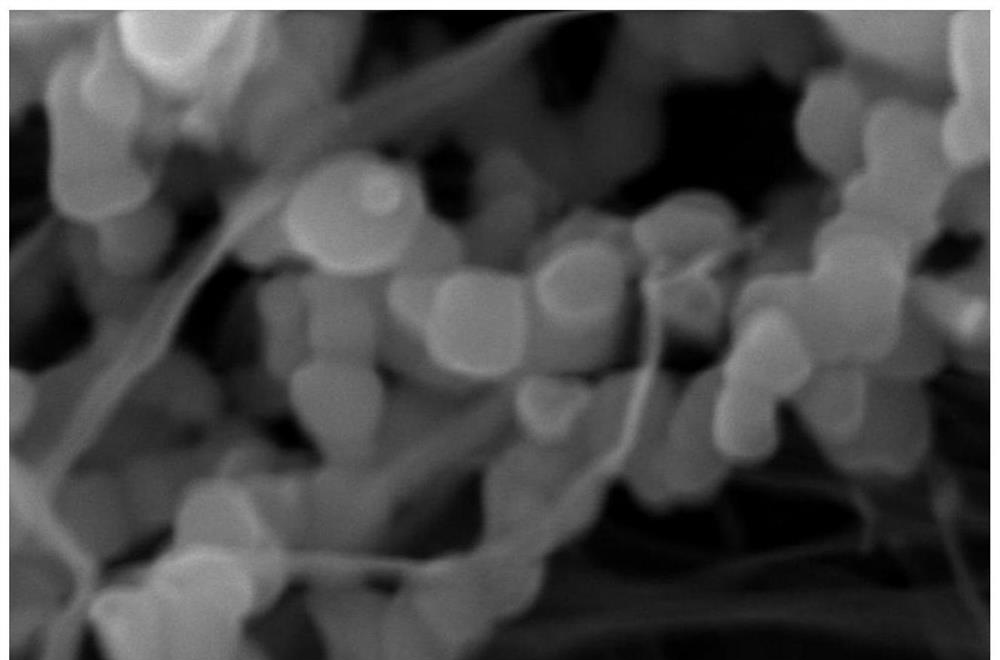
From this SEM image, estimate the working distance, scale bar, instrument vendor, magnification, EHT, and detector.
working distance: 7.1 mm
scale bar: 100 nm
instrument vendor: Zeiss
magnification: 100 K X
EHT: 15 kV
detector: InlensDuo